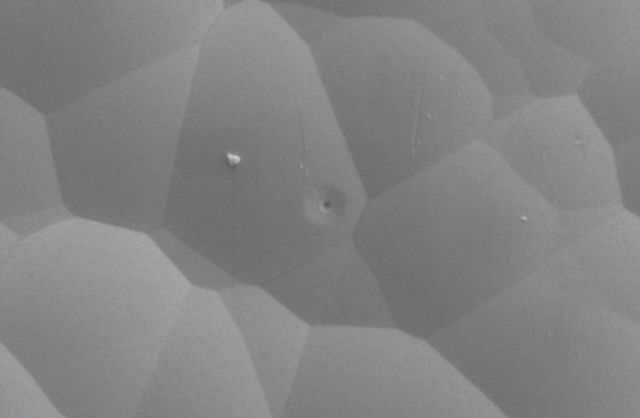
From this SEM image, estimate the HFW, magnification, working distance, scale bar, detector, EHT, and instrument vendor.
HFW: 12.7 µm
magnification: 10 K X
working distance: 3.9 mm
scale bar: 5000 nm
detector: ETD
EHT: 3 kV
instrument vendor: FEI